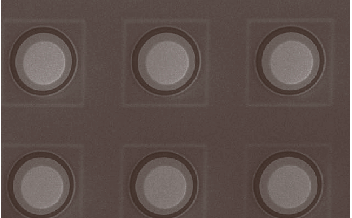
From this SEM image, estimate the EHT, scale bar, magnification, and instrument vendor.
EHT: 20 kV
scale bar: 50000 nm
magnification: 0.3 K X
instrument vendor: JEOL